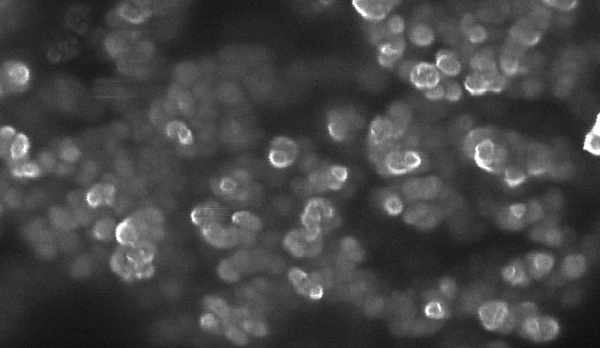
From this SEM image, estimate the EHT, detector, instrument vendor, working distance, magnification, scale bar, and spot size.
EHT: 15 kV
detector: TLD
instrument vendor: FEI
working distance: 4.8 mm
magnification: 75 K X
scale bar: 200 nm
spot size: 3